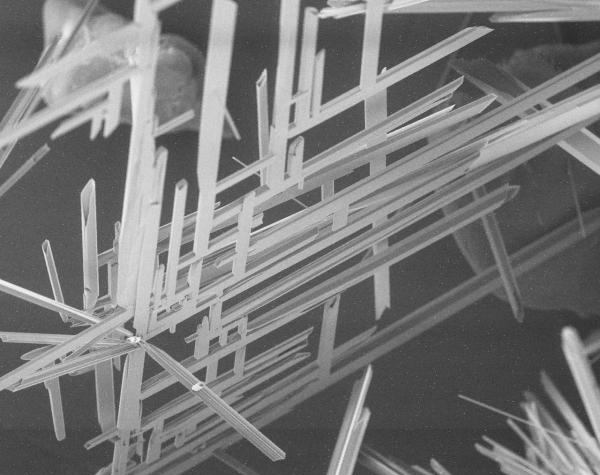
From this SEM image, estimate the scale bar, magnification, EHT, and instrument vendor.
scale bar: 200000 nm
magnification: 0.1 K X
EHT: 10 kV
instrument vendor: JEOL